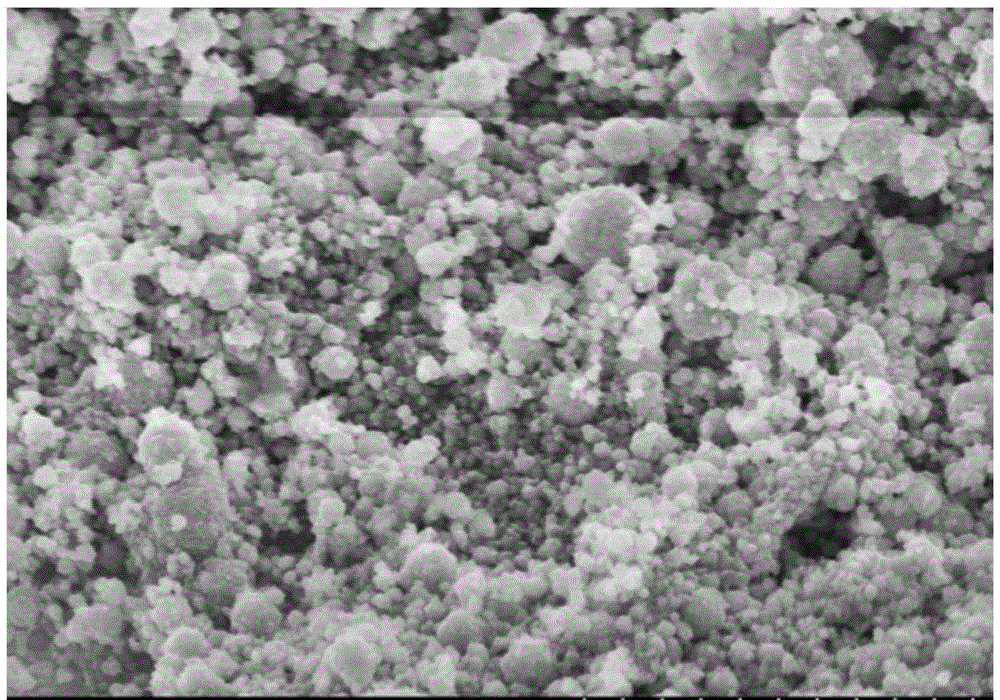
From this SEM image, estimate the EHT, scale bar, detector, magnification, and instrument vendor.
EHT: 5 kV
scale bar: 2000 nm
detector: SE(M)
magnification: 25 K X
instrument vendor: Hitachi